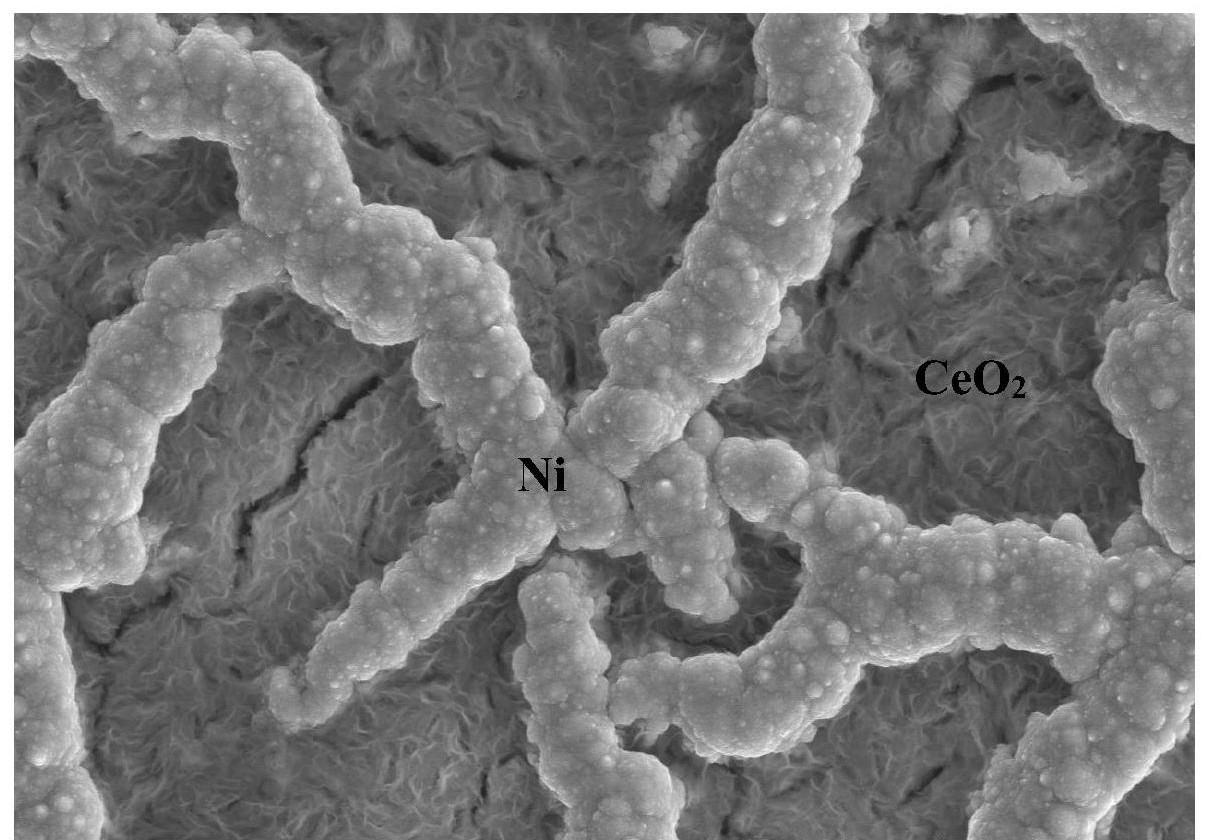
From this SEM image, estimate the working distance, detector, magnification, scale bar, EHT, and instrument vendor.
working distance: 15.7 mm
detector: SE(M)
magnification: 5 K X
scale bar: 10000 nm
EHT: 15 kV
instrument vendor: Hitachi